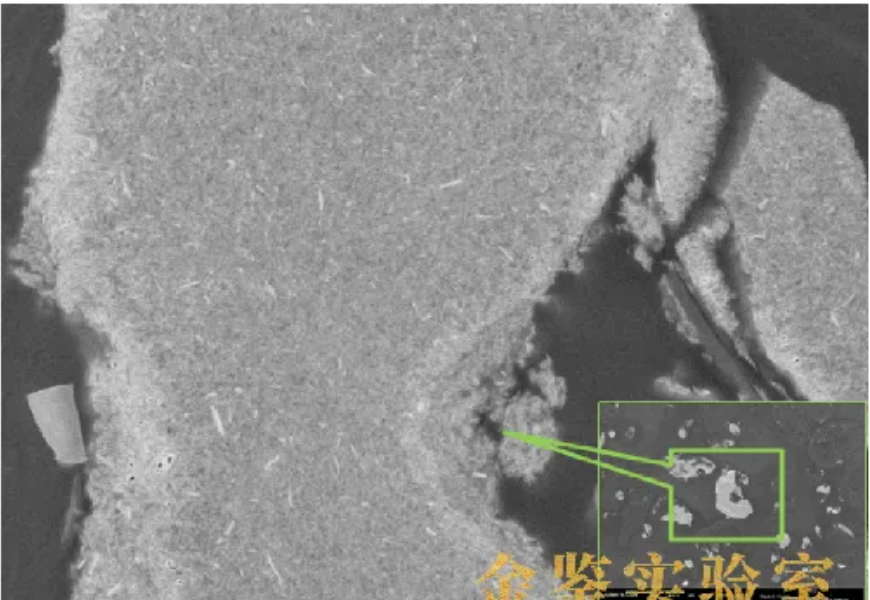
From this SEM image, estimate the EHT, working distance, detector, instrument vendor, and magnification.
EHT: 5 kV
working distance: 3.3 mm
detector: InLens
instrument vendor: Zeiss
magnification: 10 K X